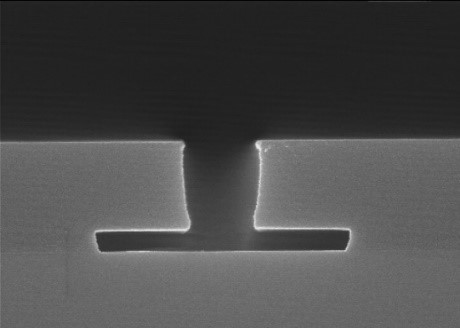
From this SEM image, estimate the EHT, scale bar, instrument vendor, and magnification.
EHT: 15 kV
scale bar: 1000 nm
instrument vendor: JEOL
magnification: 30 K X